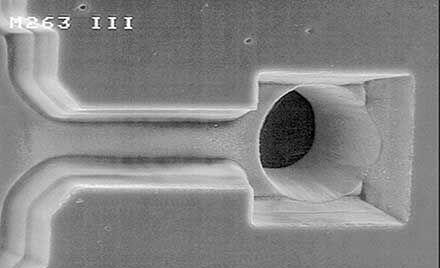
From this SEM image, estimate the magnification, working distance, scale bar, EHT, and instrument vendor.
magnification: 0.75 K X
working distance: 39 mm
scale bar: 10000 nm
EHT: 15 kV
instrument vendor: JEOL